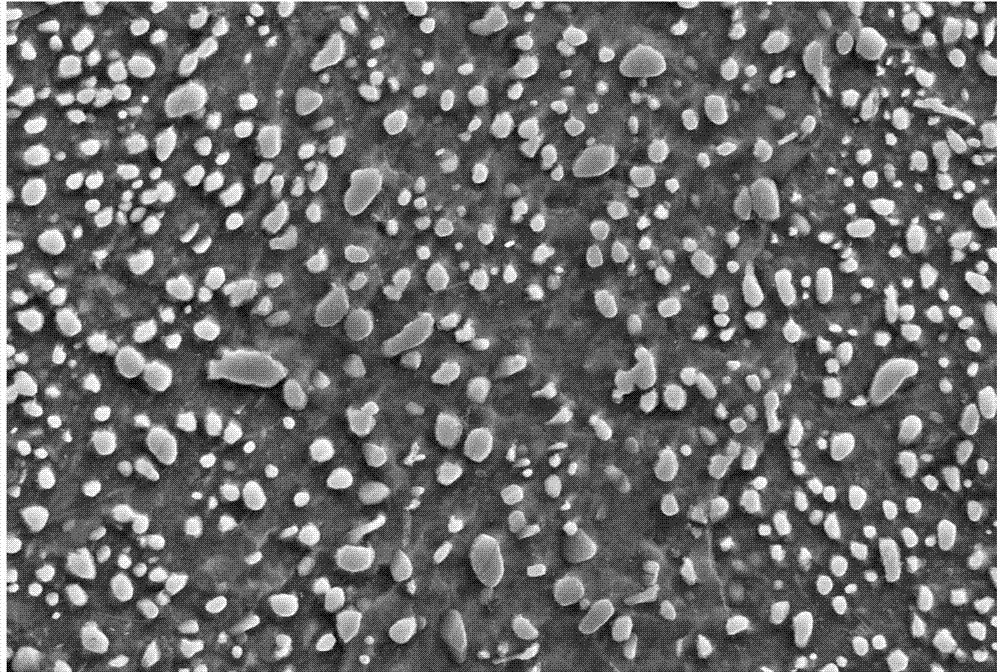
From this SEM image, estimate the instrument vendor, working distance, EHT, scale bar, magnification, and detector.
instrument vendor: Zeiss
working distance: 12.3 mm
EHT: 15 kV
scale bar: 1000 nm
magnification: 5 K X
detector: SE2